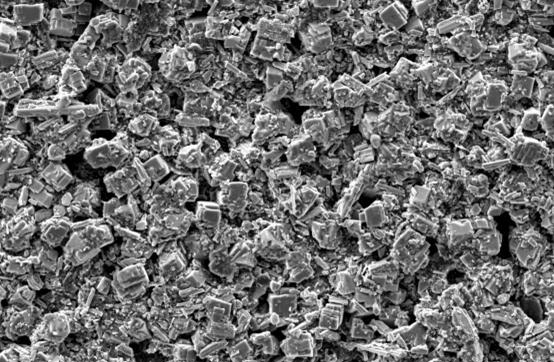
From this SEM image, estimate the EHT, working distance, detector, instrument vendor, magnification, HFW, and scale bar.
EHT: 7 kV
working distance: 6.2 mm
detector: ETD SE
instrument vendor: other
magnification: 2 K X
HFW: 64.06 µm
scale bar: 5000 nm